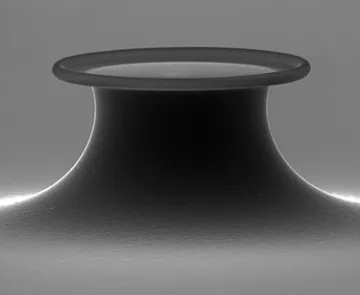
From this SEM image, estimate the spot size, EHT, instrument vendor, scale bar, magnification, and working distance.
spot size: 3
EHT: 30 kV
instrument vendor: FEI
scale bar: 50000 nm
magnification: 1.7 K X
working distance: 16.7 mm